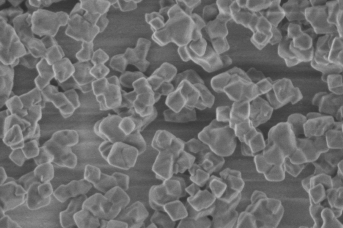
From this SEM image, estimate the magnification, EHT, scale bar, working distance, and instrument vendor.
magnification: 40 K X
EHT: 1 kV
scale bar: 1000 nm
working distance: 4 mm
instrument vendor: Hitachi